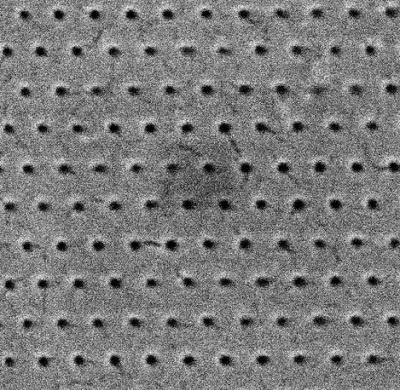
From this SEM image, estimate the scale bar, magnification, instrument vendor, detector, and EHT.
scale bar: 2000 nm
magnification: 50 K X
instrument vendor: Tescan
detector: SE Detector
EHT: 30 kV